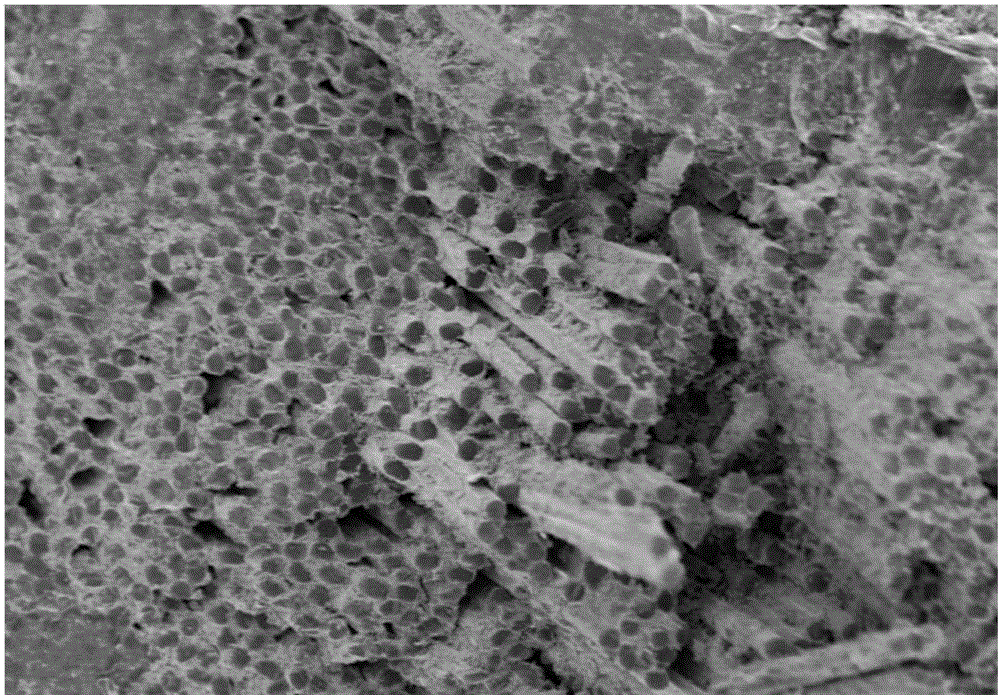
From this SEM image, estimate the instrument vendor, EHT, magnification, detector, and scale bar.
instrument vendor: Hitachi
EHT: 5 kV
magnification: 0.5 K X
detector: SE(M)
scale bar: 100000 nm